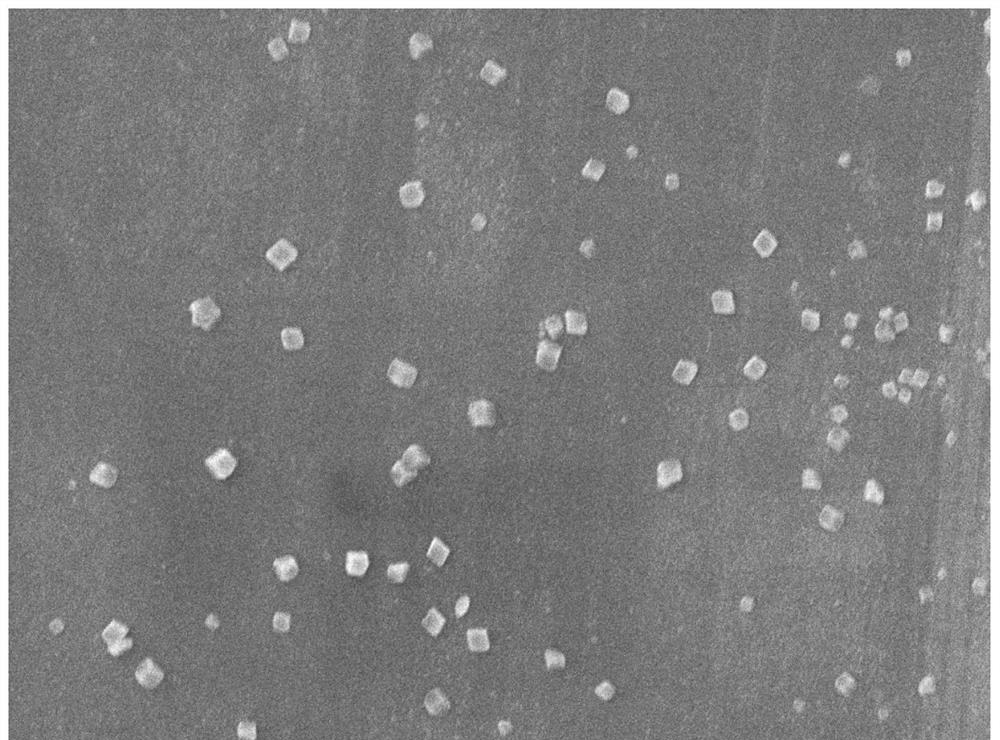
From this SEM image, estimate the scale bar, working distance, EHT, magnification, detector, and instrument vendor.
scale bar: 1000 nm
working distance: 7.9 mm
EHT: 5 kV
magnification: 10 K X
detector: SEI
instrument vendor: JEOL